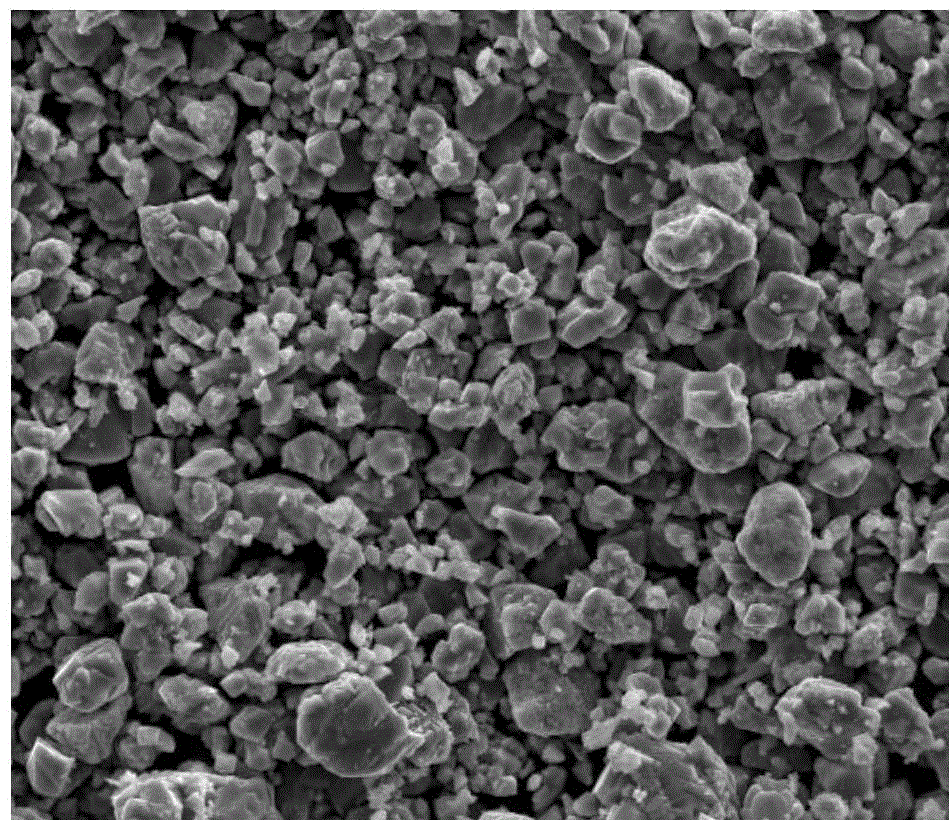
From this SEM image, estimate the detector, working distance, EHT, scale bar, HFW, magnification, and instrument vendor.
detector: ETD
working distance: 10.8 mm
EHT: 20 kV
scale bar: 50000 nm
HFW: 128 µm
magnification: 2 K X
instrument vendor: FEI